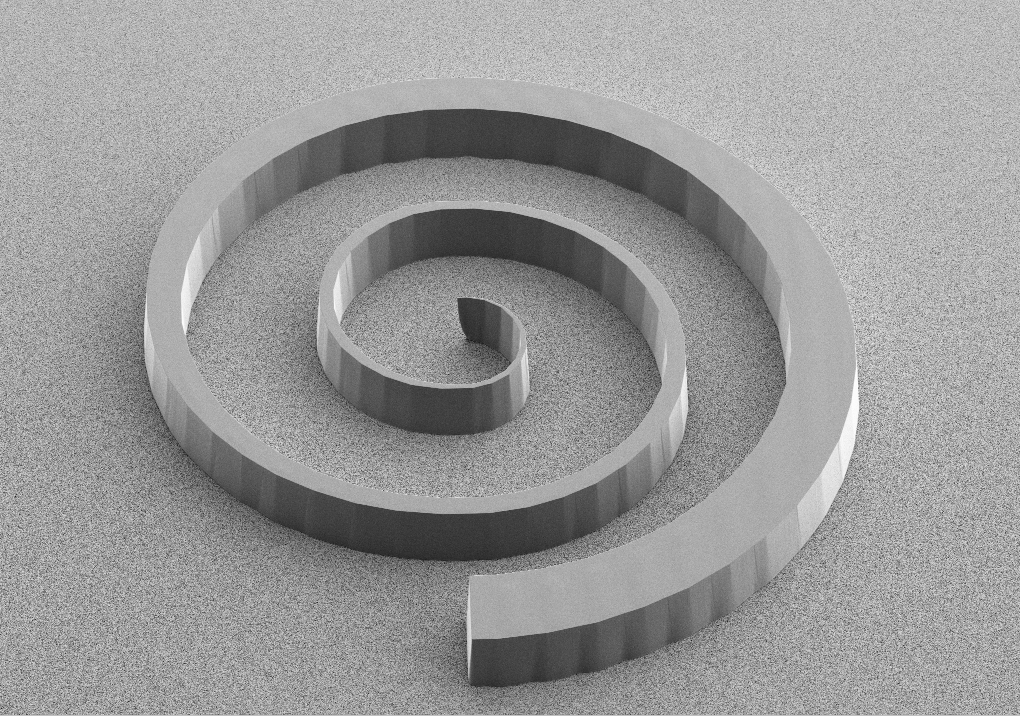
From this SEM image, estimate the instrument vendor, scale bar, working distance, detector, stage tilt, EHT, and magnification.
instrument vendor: Zeiss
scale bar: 20000 nm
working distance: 14 mm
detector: SE2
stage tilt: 45°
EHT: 5 kV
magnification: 0.1 K X